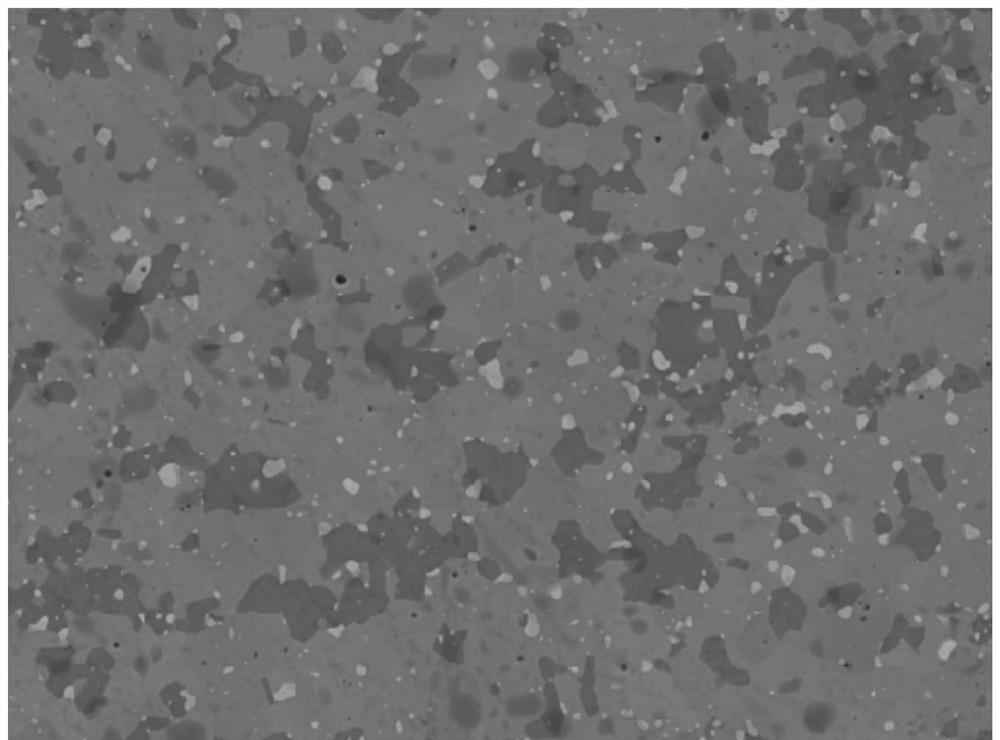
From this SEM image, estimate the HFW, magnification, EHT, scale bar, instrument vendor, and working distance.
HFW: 92.3 µm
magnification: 3 K X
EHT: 10 kV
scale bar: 20000 nm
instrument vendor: Tescan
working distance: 10.57 mm